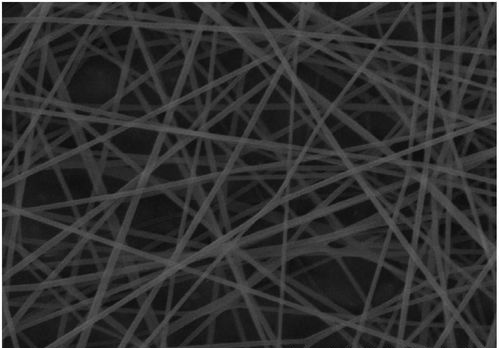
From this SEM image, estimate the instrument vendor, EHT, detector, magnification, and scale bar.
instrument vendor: Hitachi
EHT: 15 kV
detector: SE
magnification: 5 K X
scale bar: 10000 nm